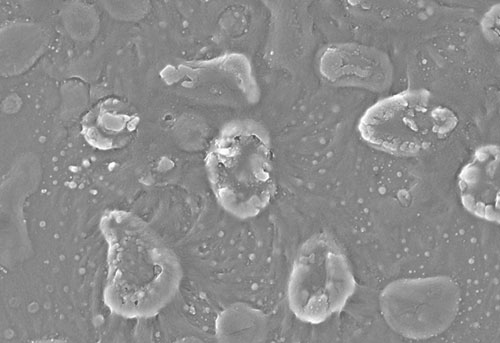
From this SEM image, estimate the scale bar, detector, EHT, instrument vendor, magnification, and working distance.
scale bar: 1000 nm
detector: InLens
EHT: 5 kV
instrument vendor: Zeiss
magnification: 25 K X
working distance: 3 mm